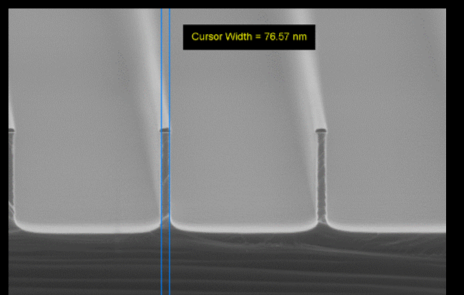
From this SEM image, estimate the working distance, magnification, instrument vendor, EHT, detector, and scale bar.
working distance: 9.2 mm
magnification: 27.71 K X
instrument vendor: Zeiss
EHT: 20 kV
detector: InLens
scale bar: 200 nm